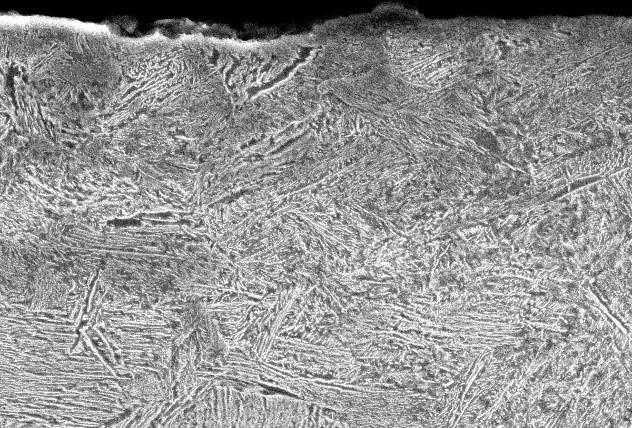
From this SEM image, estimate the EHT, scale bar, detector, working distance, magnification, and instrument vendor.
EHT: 20 kV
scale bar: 5000 nm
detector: SEI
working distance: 10 mm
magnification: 3 K X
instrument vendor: JEOL